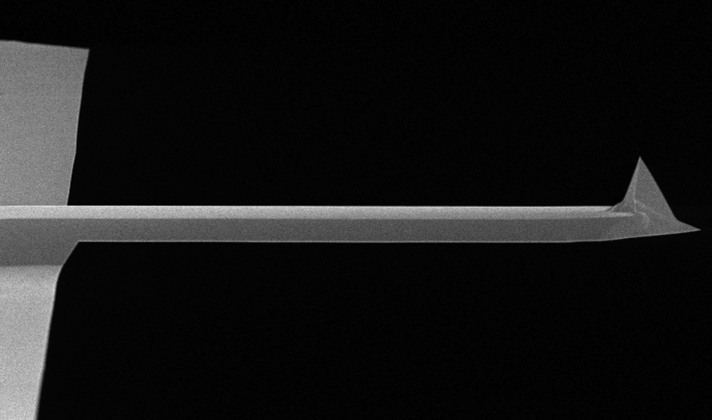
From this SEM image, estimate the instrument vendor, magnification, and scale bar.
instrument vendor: FEI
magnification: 0.85 K X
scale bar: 20000 nm